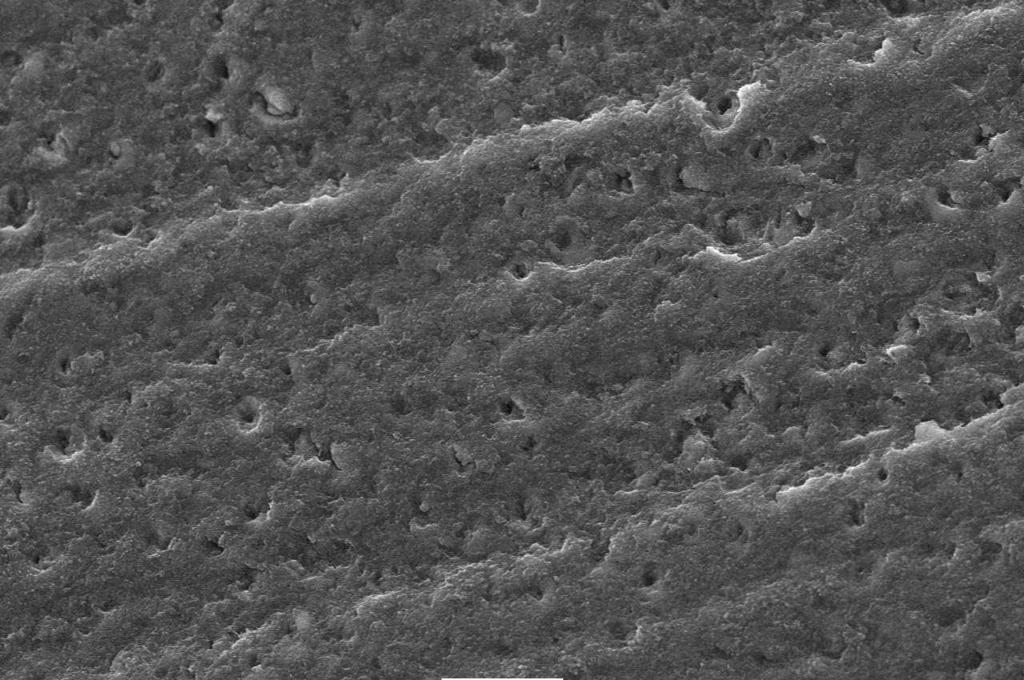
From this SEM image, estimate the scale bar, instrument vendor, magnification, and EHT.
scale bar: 10000 nm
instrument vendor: JEOL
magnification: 1.5 K X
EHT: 15 kV